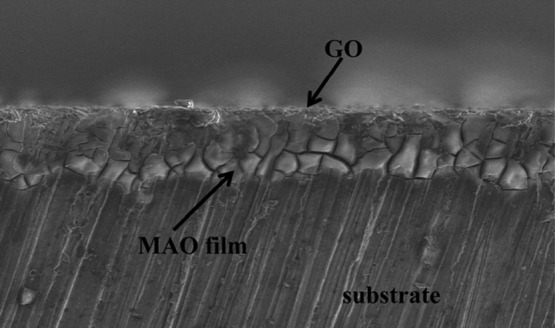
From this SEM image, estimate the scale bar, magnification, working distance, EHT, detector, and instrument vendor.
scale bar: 50000 nm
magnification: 1 K X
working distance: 6.6 mm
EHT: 3 kV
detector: SE(L)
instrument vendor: Hitachi